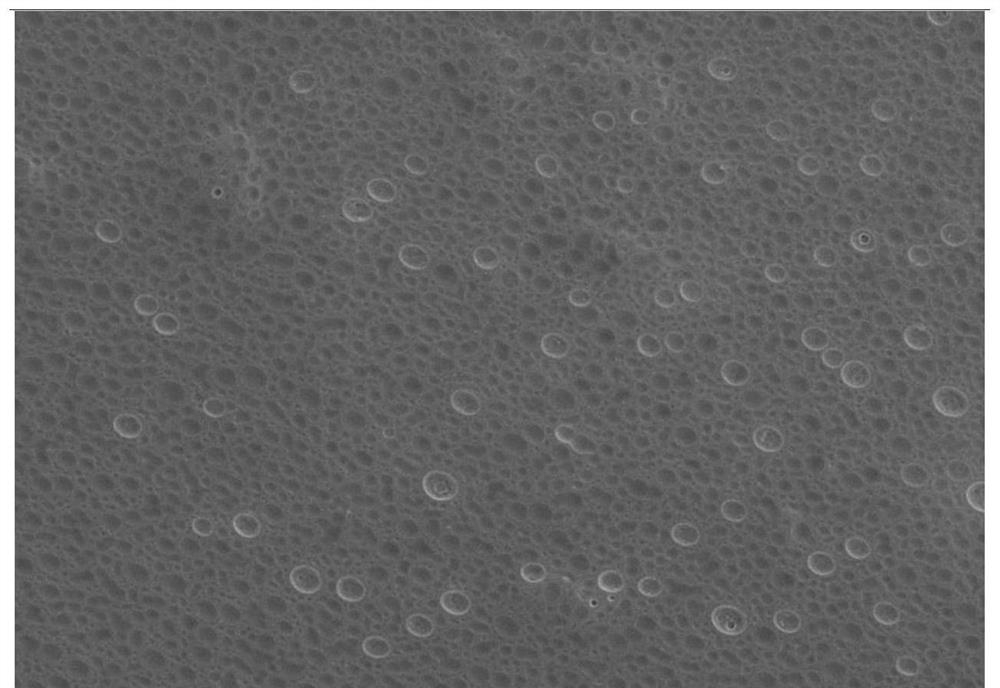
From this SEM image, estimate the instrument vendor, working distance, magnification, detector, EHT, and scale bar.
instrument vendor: Hitachi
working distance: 15 mm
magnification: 1.5 K X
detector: SE(UL)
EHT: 3 kV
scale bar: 30000 nm